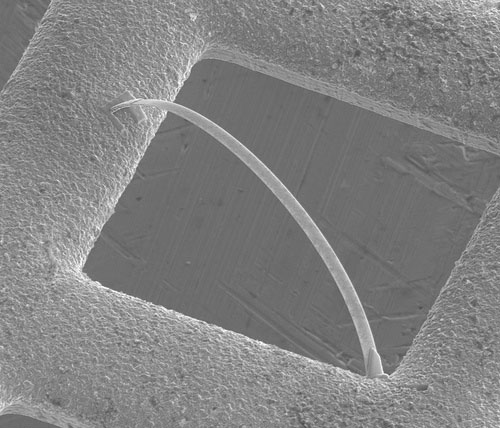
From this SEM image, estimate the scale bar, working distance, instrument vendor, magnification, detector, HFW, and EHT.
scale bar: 20000 nm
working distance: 5.8 mm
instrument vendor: FEI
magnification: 2 K X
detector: ETD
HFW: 64 µm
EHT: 5 kV